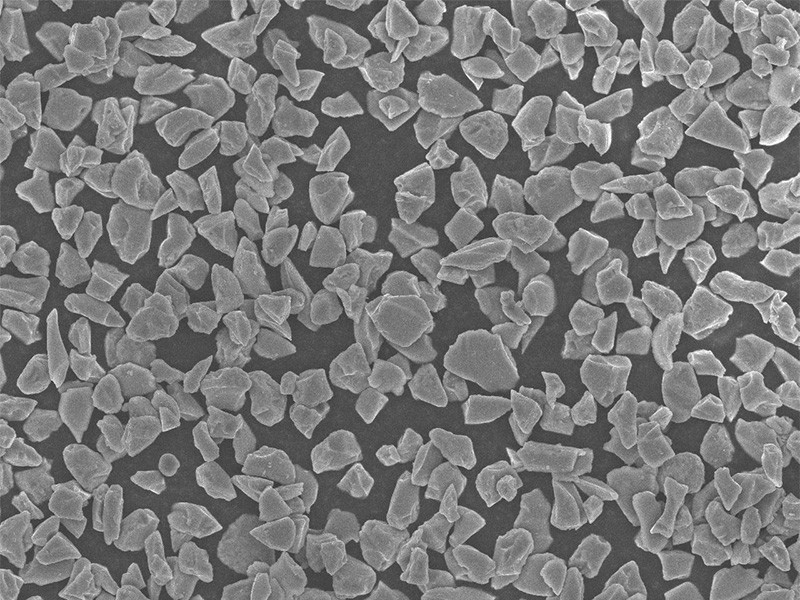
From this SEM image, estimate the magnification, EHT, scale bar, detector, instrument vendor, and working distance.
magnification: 1 K X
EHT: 10 kV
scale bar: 10000 nm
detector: SEI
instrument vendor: JEOL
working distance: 7.6 mm